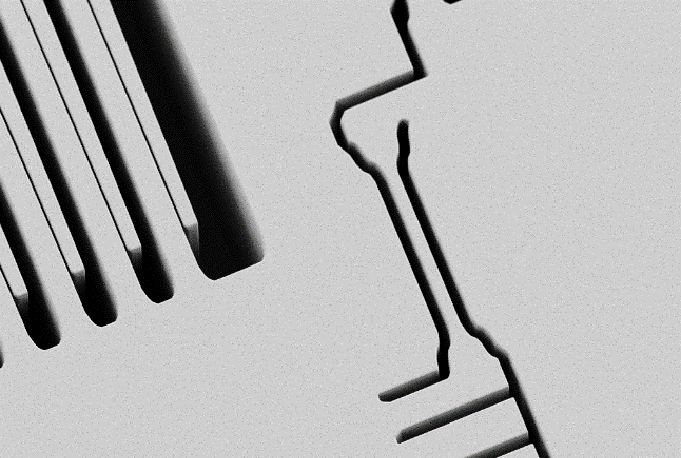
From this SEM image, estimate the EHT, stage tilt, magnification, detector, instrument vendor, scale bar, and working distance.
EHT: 1 kV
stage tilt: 25°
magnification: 0.5 K X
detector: SE2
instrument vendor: Zeiss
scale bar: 20000 nm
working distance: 6.1 mm